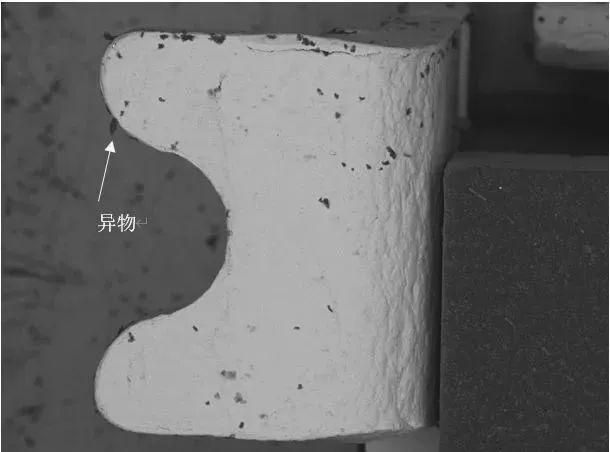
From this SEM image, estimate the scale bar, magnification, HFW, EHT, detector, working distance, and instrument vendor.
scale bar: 500000 nm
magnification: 0.127 K X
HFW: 2180 µm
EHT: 20 kV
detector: BSE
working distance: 13.59 mm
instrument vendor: Tescan